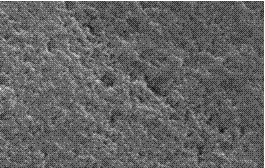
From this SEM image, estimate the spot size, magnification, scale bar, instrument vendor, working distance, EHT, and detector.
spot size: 4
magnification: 50 K X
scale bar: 1000 nm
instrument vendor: FEI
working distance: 6.4 mm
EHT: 25 kV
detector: SE